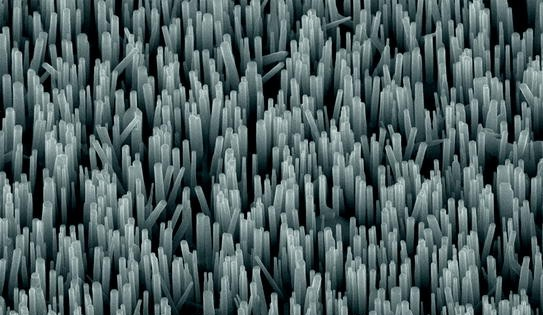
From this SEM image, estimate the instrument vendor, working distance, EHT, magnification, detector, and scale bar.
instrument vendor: Zeiss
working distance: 5 mm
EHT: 20 kV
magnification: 20.63 K X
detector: InLens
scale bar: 2000 nm